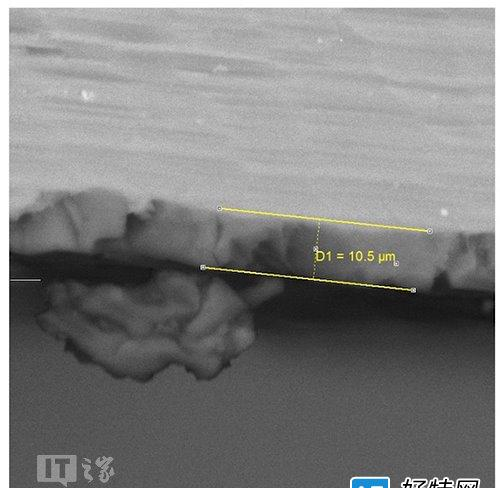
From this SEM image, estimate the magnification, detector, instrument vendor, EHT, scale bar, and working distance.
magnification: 2.34 K X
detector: BSE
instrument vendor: Tescan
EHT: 25 kV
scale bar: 20000 nm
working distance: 25.94 mm